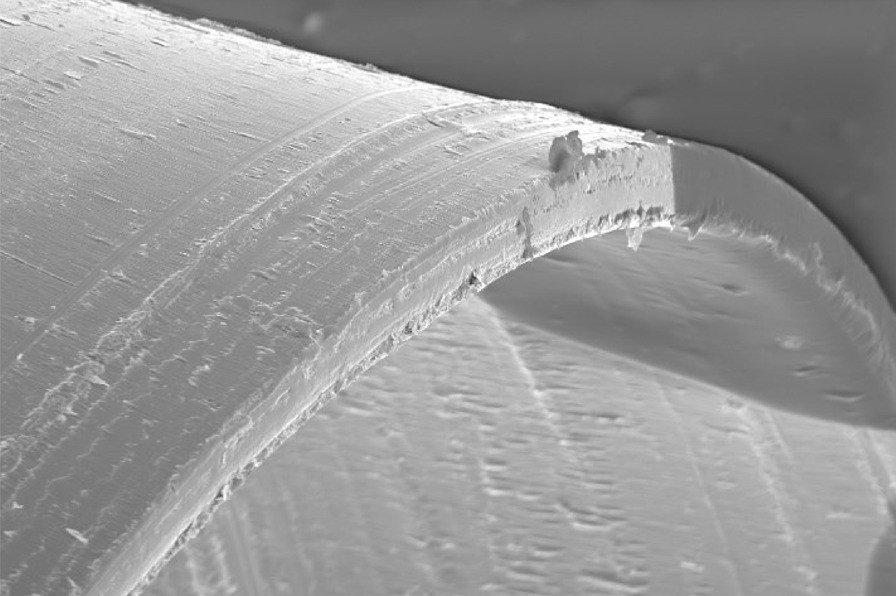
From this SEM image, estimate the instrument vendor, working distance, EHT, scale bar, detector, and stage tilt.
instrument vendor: FEI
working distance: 18.4 mm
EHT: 20 kV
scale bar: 100000 nm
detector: SE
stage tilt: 0°